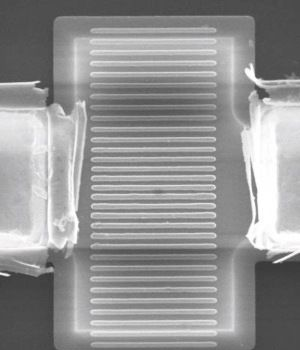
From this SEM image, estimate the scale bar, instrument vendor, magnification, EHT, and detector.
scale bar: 1000 nm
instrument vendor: JEOL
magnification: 8 K X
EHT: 15 kV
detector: SEI